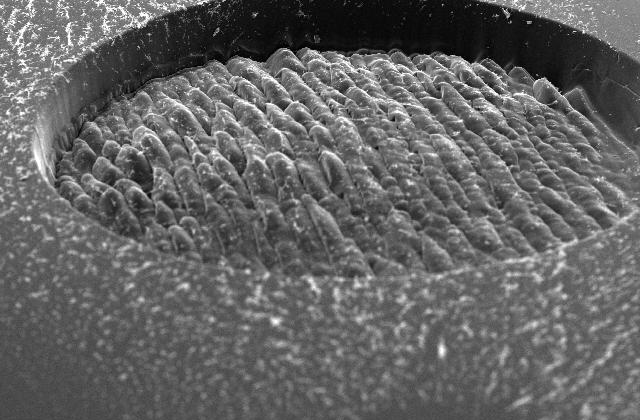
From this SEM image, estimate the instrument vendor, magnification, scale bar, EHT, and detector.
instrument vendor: JEOL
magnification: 0.06 K X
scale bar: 200000 nm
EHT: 20 kV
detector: SEI